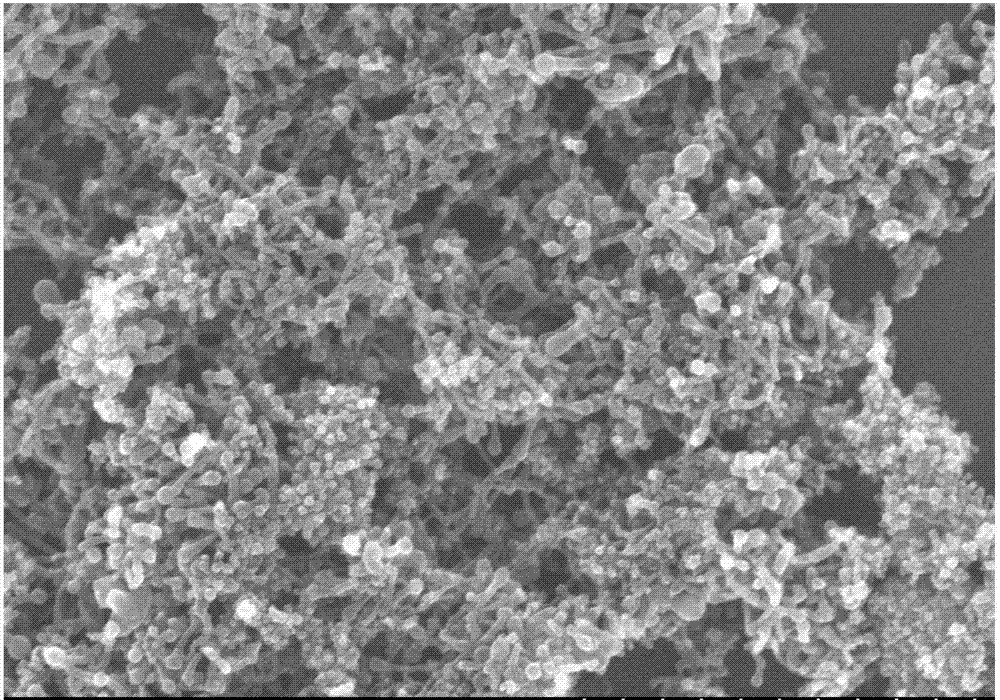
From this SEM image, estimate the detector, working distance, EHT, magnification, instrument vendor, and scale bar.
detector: SE(M)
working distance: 8.5 mm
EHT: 15 kV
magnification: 50 K X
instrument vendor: Hitachi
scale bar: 1000 nm